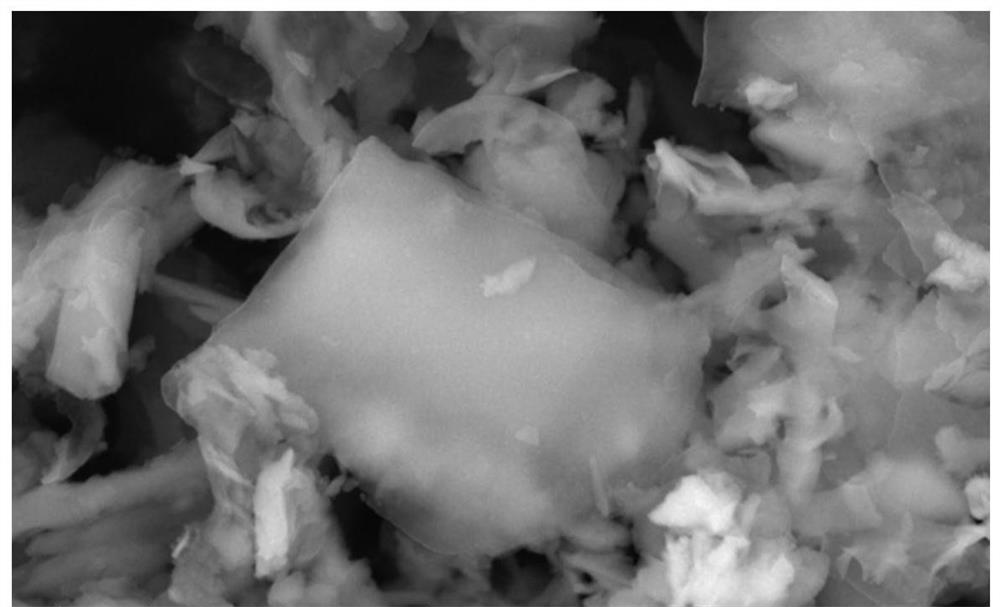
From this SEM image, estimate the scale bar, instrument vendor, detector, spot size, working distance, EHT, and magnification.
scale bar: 1000 nm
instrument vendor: FEI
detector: TLD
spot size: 3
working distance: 5 mm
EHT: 15 kV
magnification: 100 K X